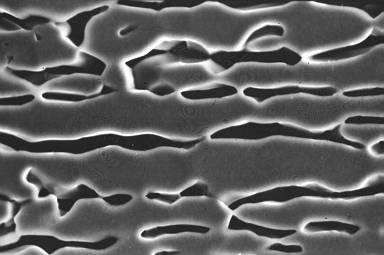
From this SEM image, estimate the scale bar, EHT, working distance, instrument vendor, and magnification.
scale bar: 1000 nm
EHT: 15 kV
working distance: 15 mm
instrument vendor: JEOL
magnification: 4.5 K X